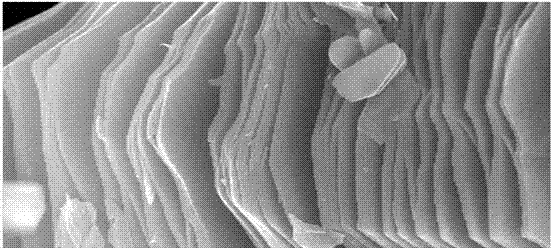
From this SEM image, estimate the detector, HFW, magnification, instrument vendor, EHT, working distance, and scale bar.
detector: SE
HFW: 4.14 µm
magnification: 50 K X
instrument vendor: FEI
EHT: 15 kV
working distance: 6.4 mm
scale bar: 1000 nm